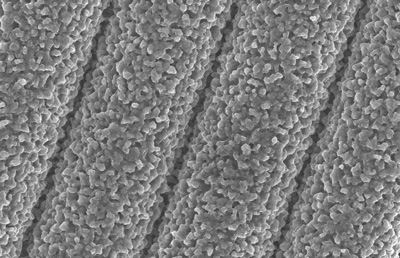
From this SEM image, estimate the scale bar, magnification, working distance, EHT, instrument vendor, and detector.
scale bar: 1000 nm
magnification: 5 K X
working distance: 4.8 mm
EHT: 8 kV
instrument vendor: Zeiss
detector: InLens